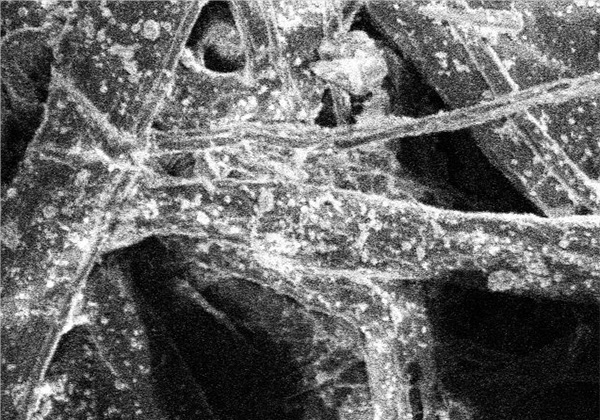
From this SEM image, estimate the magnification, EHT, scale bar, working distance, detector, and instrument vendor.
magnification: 1 K X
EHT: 10 kV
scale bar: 50000 nm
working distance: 25.4 mm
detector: SE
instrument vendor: Hitachi